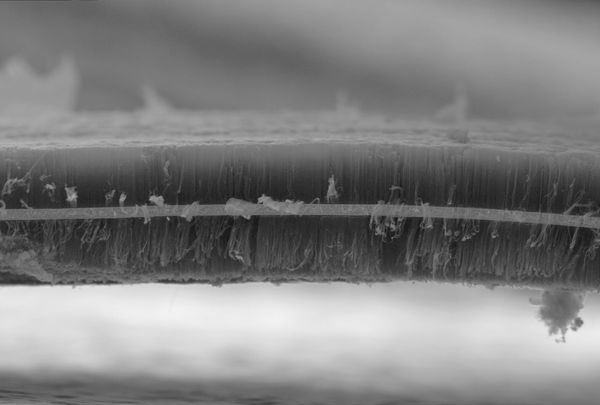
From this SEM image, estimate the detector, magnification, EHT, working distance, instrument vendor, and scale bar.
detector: SE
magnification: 0.2 K X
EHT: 25 kV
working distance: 13.6 mm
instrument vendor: JEOL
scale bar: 200000 nm